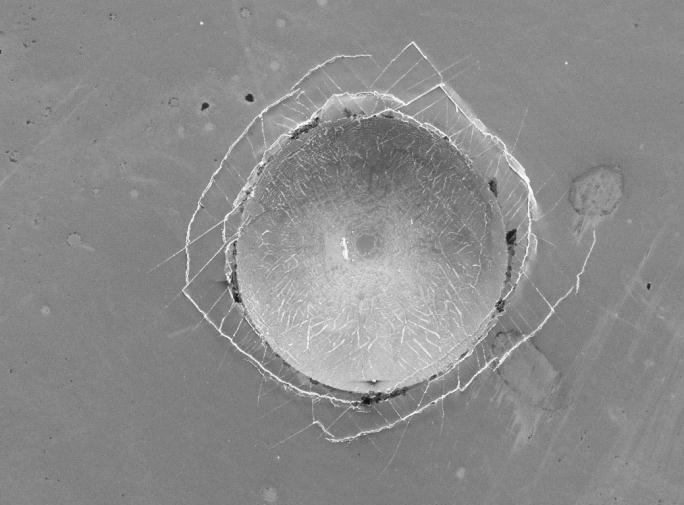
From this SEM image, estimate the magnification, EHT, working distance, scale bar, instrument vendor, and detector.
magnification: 0.05 K X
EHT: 15 kV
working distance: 38 mm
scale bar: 100000 nm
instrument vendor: JEOL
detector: SEI